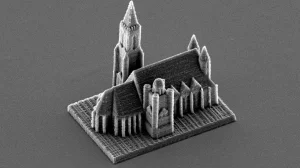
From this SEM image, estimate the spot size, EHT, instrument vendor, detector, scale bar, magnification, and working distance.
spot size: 4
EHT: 12 kV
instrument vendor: FEI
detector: SE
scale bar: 50000 nm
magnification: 0.628 K X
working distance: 20.1 mm